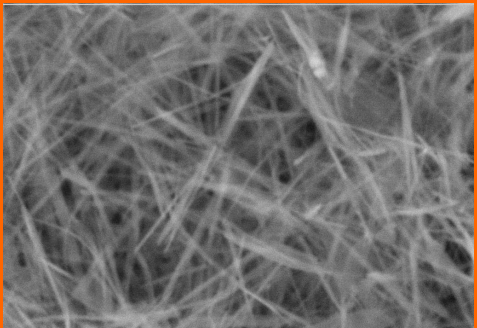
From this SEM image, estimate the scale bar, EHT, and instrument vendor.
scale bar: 1000 nm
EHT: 20 kV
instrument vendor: JEOL